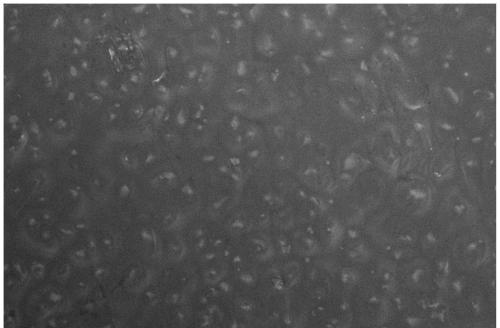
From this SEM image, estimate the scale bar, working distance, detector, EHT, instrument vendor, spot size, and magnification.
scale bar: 10000 nm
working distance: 4.9 mm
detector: TLD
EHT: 5 kV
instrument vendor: FEI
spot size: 4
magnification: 5 K X